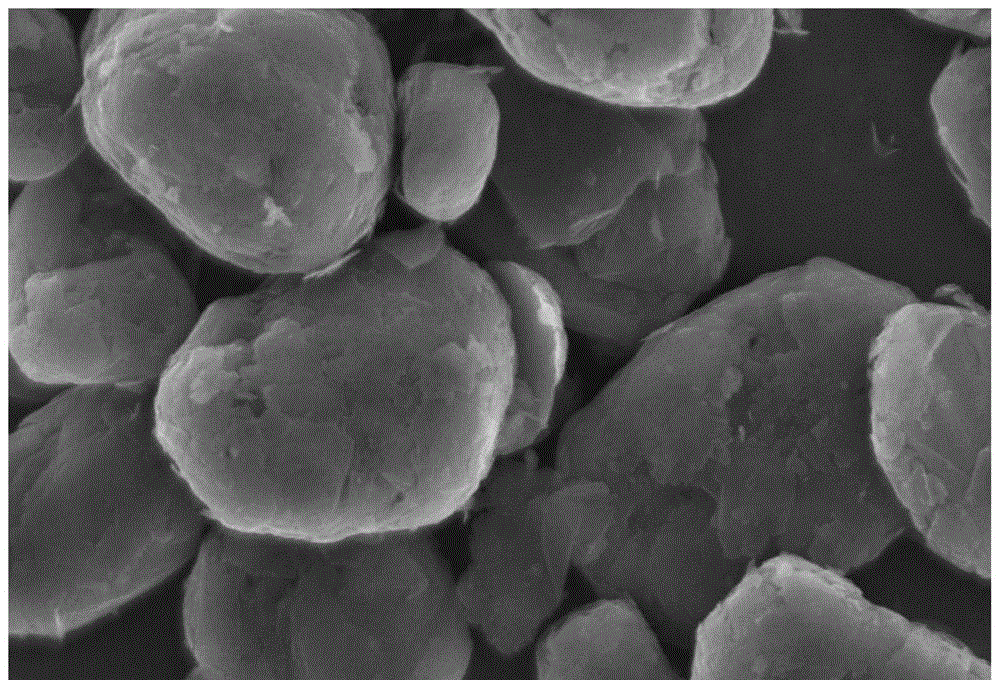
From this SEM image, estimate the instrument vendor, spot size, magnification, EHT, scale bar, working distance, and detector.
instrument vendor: JEOL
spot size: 30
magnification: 2 K X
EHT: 20 kV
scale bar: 10000 nm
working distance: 13 mm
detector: SEI